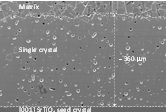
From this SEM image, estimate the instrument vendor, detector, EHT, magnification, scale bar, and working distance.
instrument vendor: Hitachi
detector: SE(U)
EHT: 15 kV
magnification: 0.2 K X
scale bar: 200000 nm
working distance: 11.7 mm